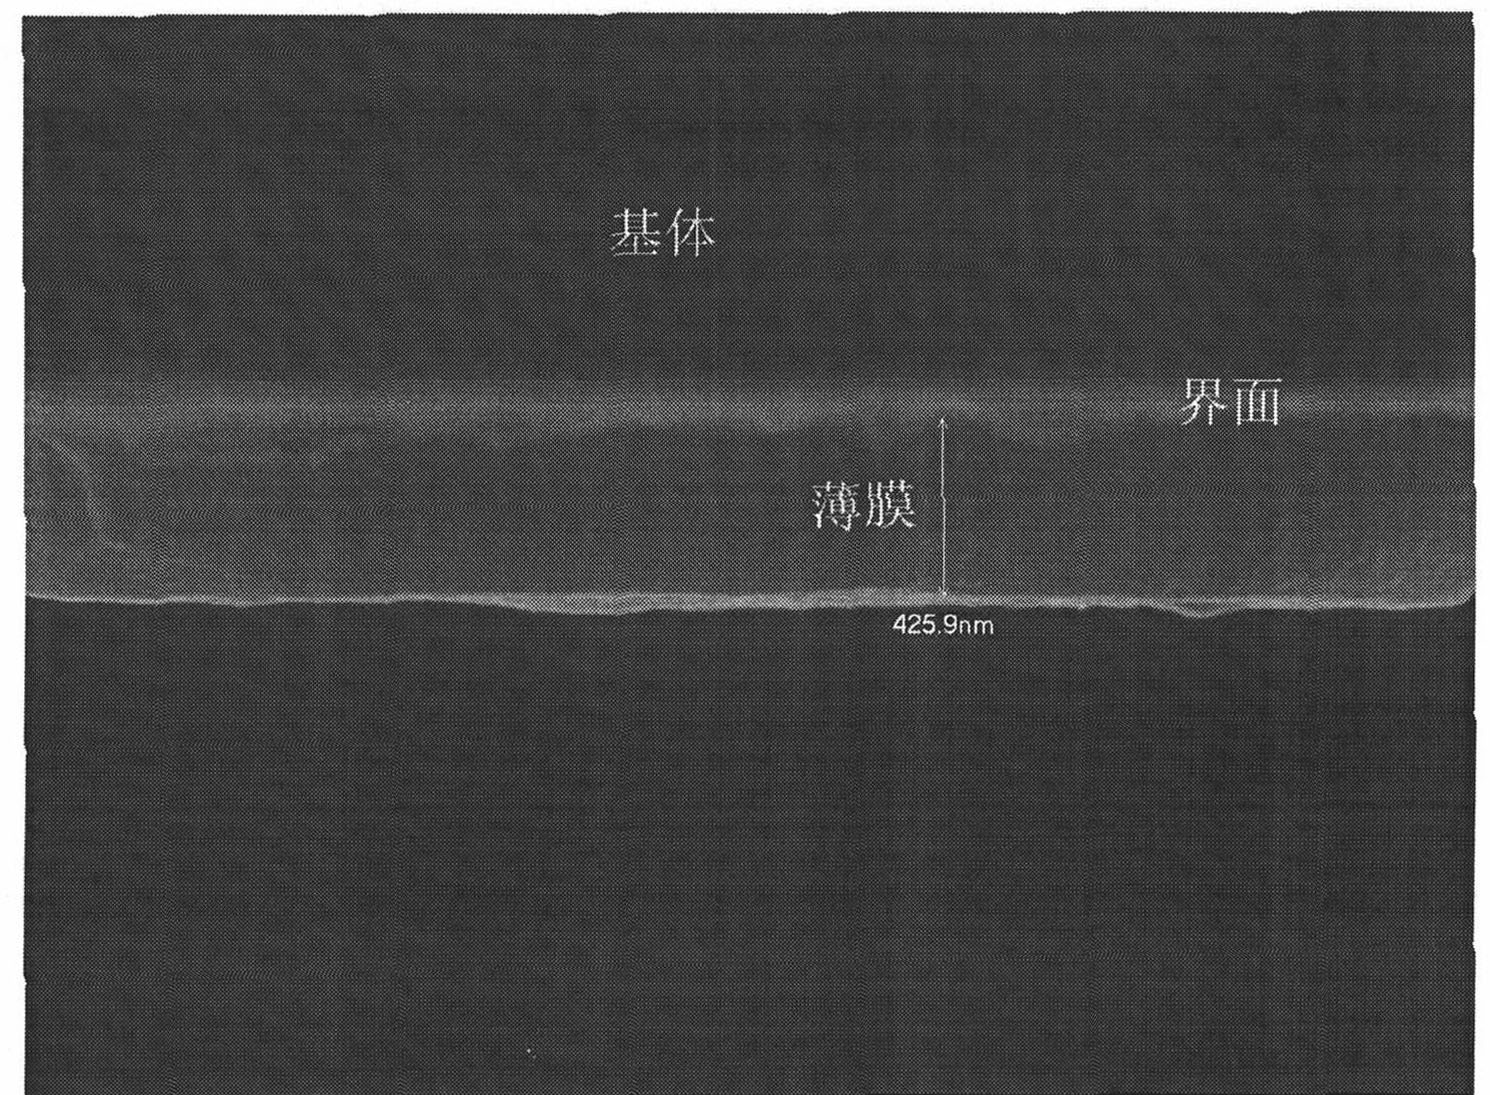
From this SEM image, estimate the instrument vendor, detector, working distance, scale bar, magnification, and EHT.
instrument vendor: JEOL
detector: SEI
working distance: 9.7 mm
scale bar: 100 nm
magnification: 35 K X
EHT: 20 kV